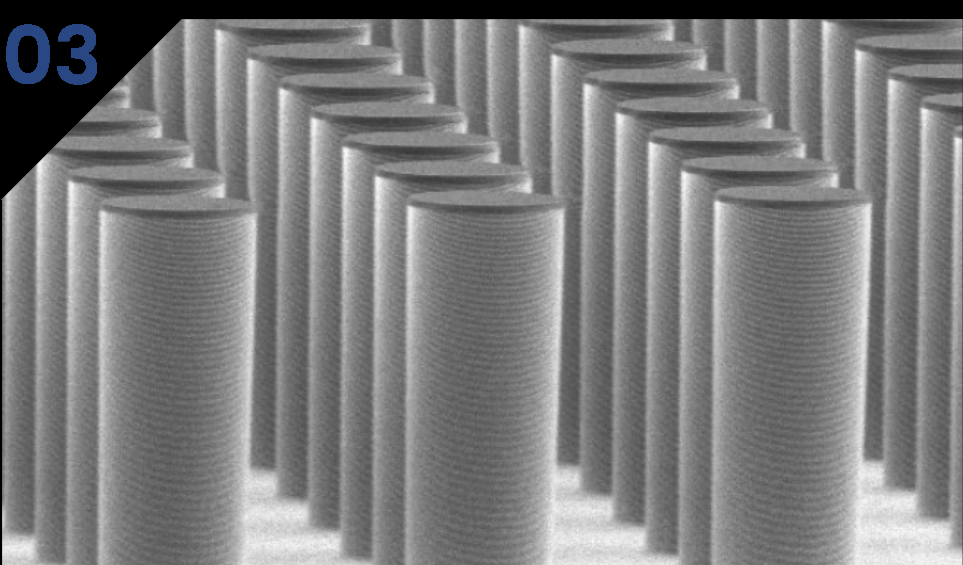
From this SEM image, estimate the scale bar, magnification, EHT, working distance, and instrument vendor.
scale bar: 100000 nm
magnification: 0.4 K X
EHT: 5 kV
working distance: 12 mm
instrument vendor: Hitachi